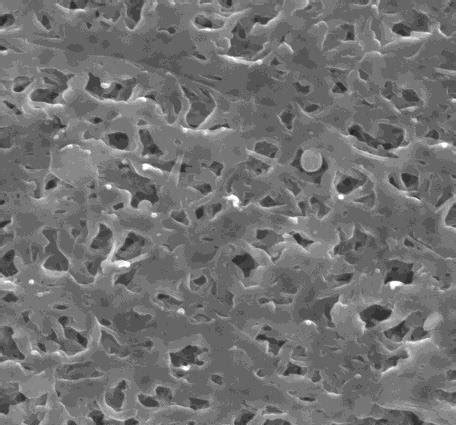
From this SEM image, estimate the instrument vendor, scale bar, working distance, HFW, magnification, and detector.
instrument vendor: FEI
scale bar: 5000 nm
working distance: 16.9 mm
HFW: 13.52 µm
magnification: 20 K X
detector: SE